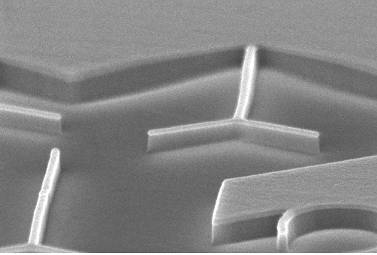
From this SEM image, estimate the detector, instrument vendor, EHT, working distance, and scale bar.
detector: InLens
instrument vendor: Zeiss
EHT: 5 kV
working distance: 4 mm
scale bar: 200 nm